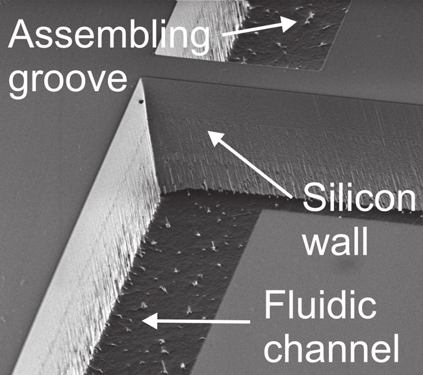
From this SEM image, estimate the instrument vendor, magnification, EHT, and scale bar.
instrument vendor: Hitachi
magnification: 0.3 K X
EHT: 4 kV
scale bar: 100000 nm